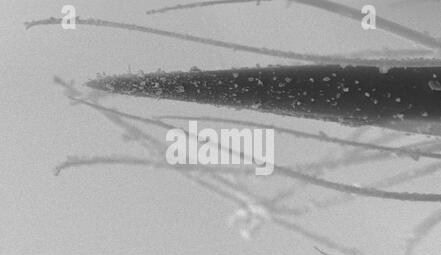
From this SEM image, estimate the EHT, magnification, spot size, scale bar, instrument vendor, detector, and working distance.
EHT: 25 kV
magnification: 0.646 K X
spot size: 3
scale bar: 50000 nm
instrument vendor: FEI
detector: SE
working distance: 5.1 mm